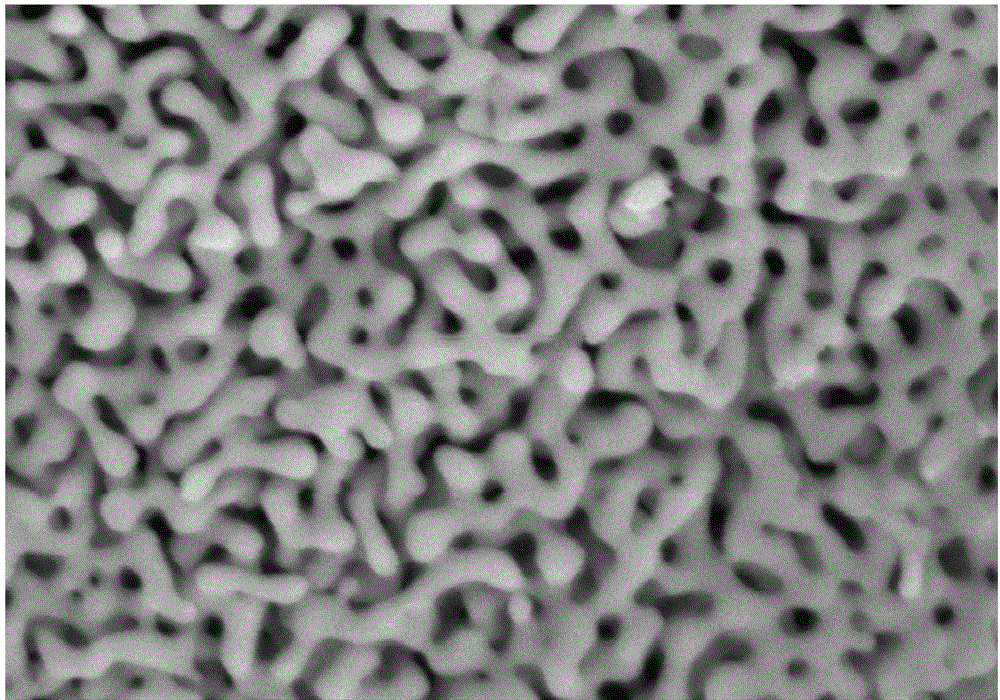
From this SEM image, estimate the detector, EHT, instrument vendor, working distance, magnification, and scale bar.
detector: SE(M,LA0)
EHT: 5 kV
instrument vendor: Hitachi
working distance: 9.2 mm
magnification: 100 K X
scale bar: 500 nm